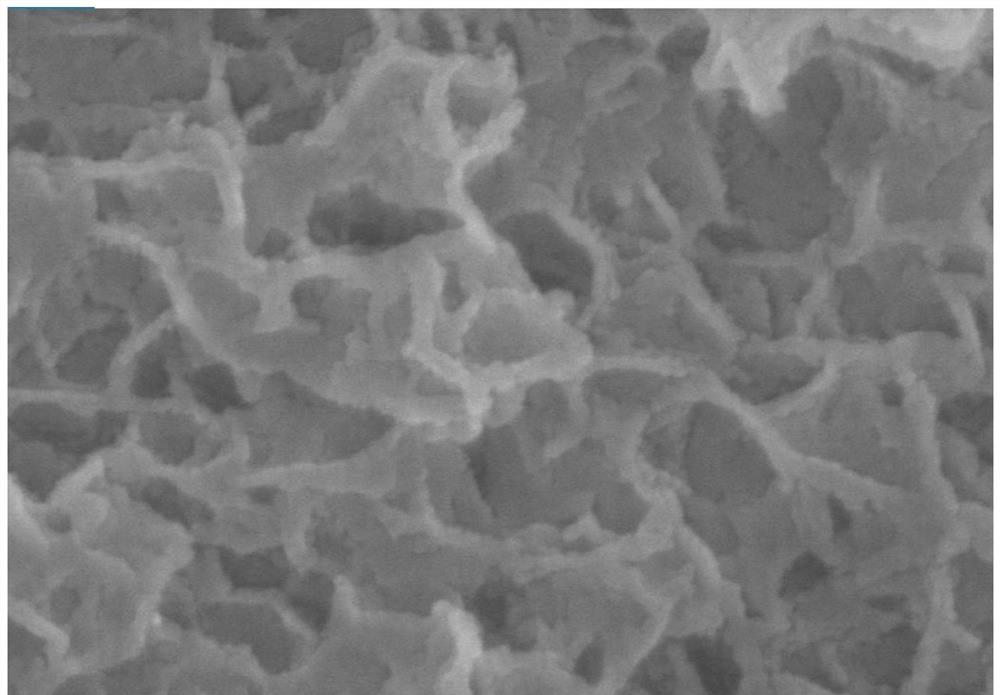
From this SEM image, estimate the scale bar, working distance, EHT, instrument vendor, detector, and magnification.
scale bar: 200 nm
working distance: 8.1 mm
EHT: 15 kV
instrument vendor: Hitachi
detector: SE(U)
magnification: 200 K X